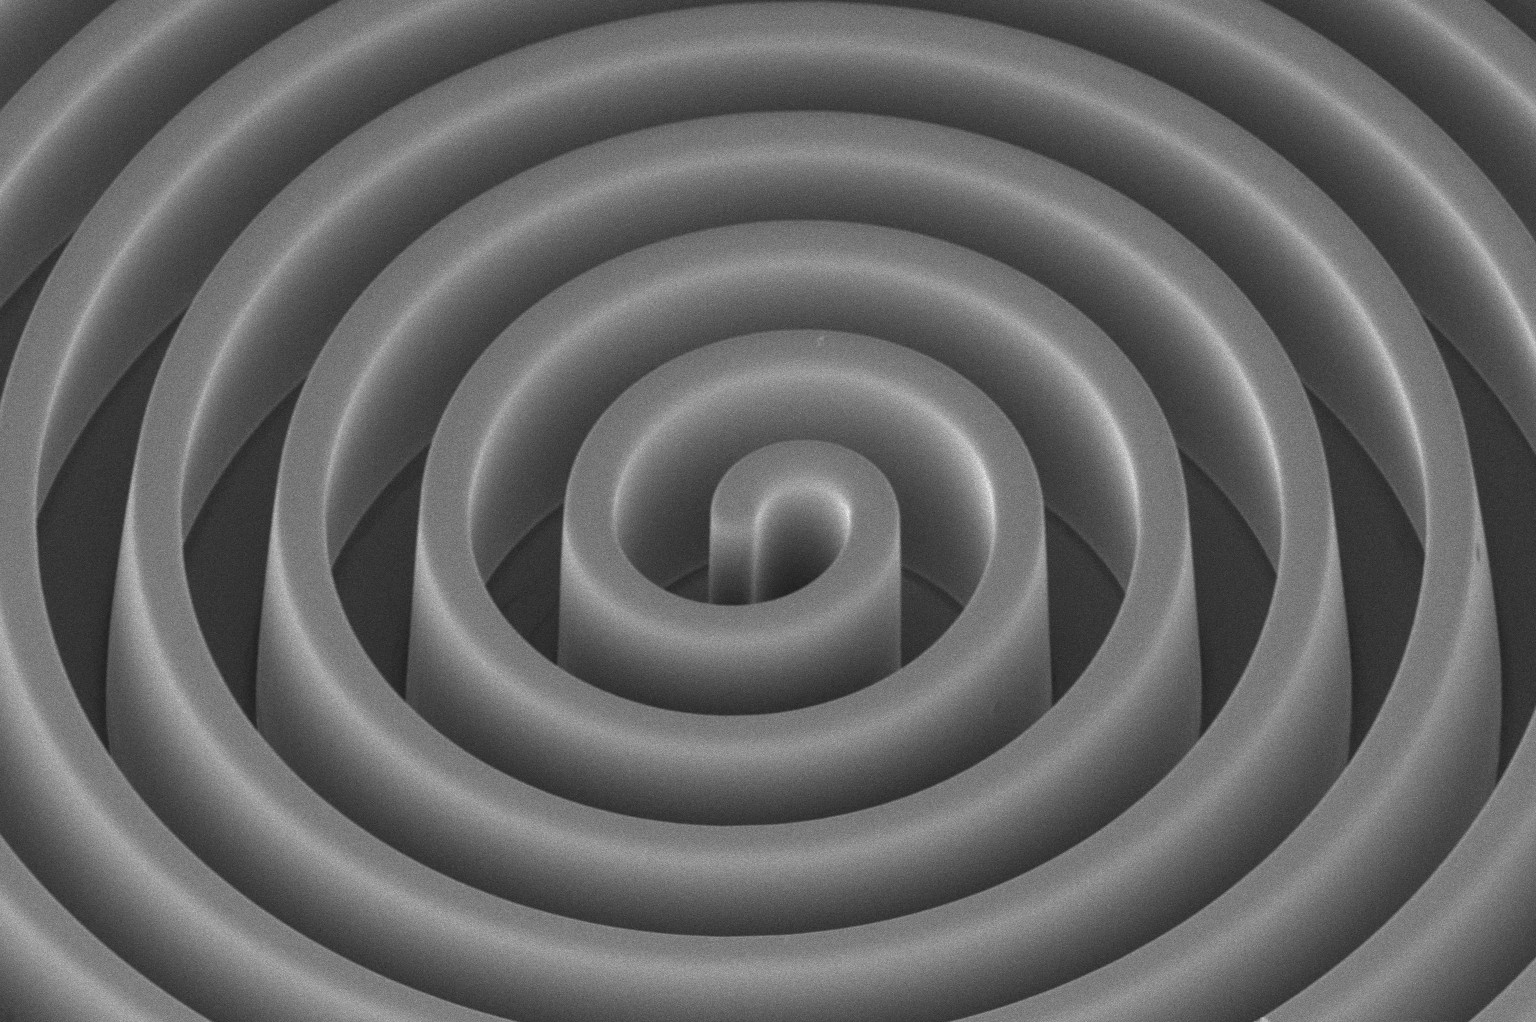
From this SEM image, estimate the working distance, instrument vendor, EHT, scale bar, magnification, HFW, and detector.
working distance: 4.1 mm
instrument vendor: FEI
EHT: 2 kV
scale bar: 50000 nm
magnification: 0.8 K X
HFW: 259 µm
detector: TLD